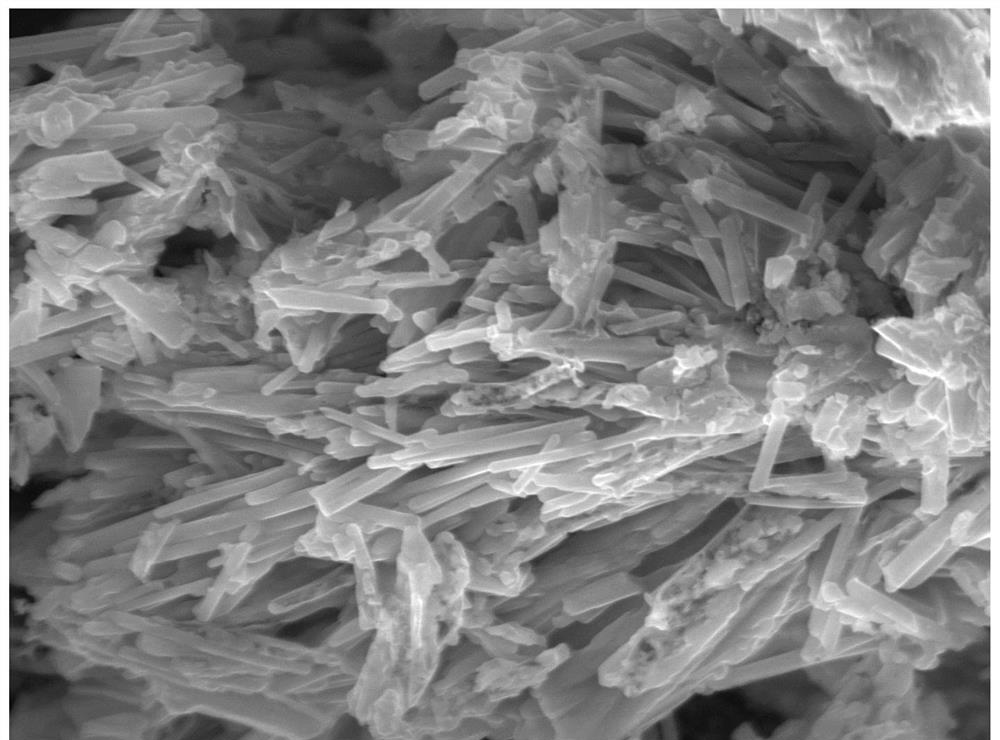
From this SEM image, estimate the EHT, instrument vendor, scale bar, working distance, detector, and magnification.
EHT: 15 kV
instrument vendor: JEOL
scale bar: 1000 nm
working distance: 10.3 mm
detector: SEI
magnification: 8 K X